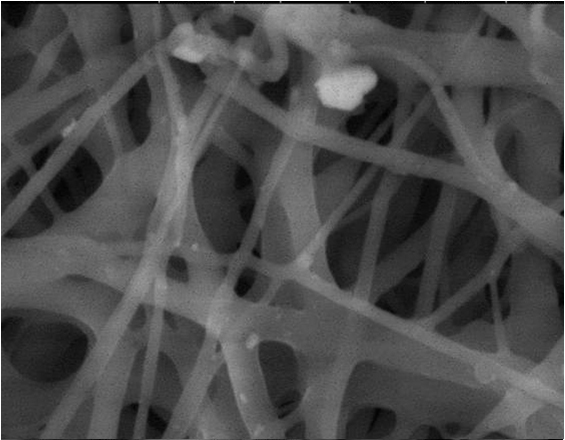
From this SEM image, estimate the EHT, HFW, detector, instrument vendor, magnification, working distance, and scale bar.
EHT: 15 kV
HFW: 29.8 µm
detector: LFD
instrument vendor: FEI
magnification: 5 K X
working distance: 9.7 mm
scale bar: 10000 nm